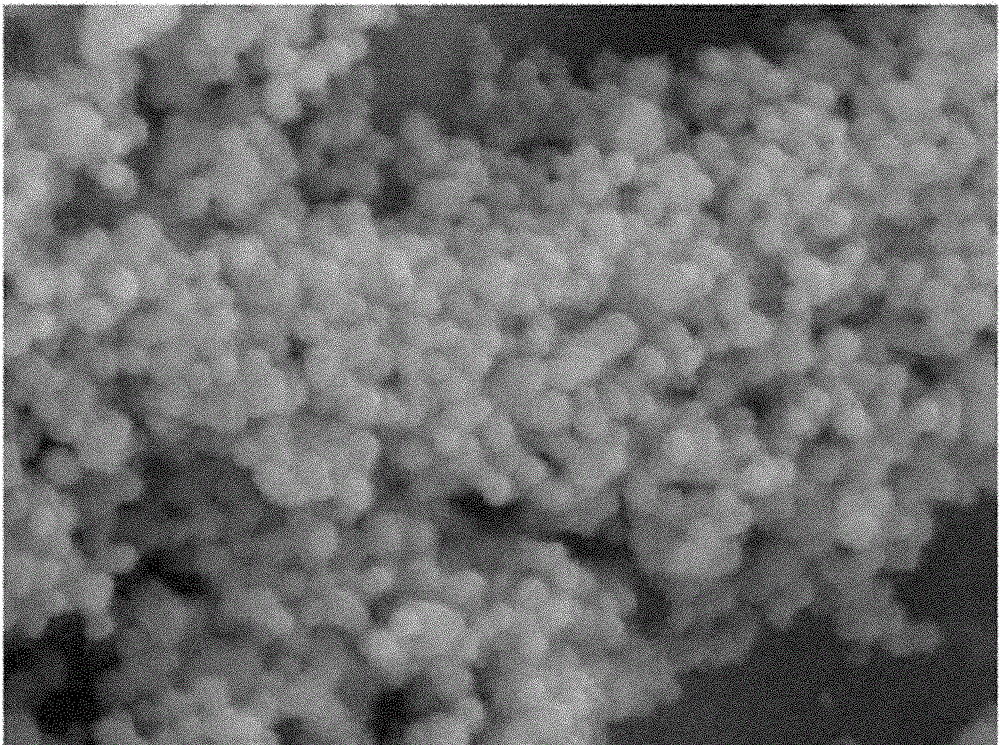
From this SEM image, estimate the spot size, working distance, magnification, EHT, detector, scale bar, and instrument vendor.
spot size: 5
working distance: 6.7 mm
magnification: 10 K X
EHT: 20 kV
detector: SE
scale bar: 2000 nm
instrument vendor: FEI